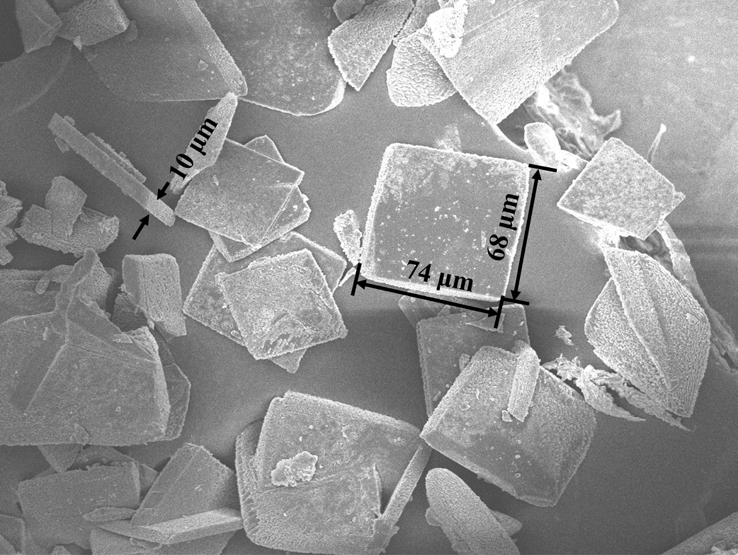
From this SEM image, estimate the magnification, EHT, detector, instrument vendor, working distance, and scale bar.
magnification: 0.3 K X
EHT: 10 kV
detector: SEI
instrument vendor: JEOL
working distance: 9.3 mm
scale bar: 10000 nm